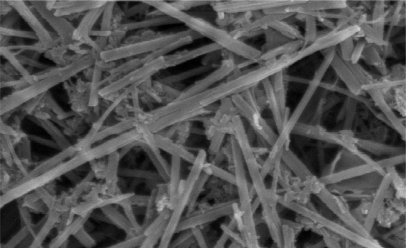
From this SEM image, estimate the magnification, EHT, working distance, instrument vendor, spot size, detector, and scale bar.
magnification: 100 K X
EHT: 25 kV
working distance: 5.8 mm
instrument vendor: FEI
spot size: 4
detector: TLD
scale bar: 500 nm